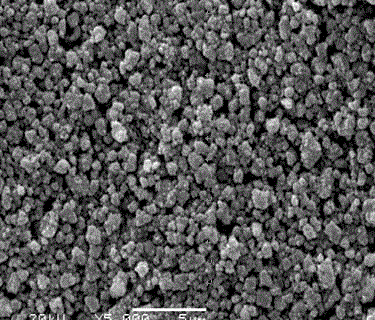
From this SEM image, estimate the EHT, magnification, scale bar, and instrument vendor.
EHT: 20 kV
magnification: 5 K X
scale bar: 5000 nm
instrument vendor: JEOL